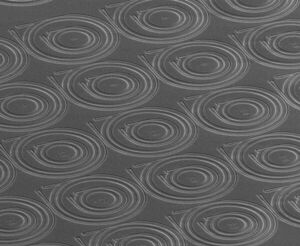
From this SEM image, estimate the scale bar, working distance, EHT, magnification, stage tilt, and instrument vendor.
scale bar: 100000 nm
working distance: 6.7 mm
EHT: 20 kV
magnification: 0.363 K X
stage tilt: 60°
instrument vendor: FEI